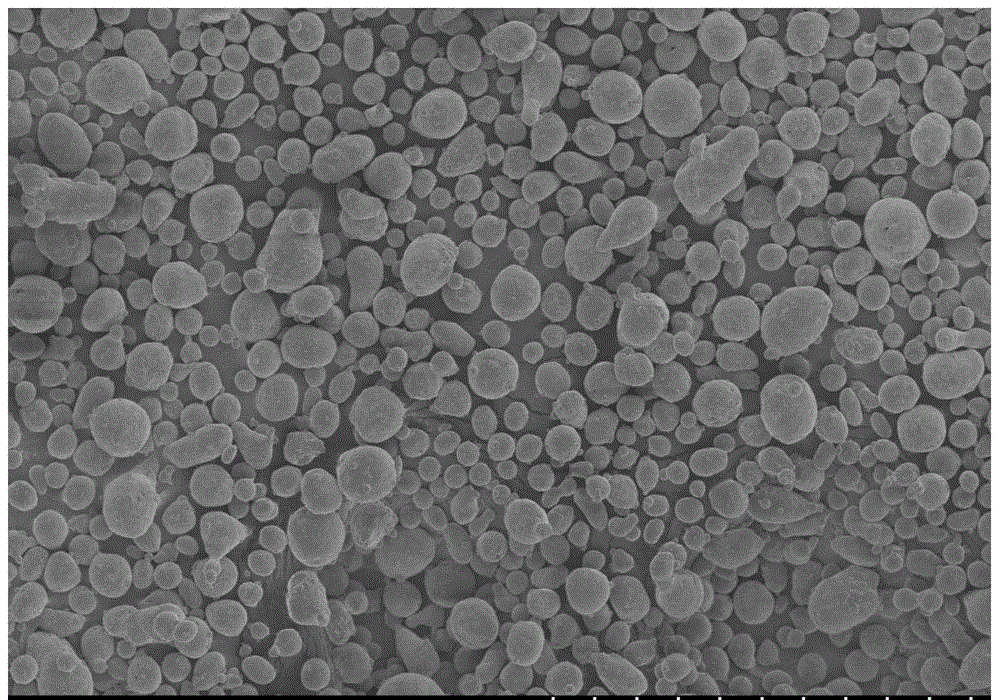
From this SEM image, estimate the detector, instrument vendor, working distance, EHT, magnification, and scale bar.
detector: SE(M)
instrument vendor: Hitachi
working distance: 15.4 mm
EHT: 5 kV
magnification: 0.18 K X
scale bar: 300000 nm